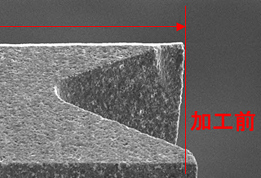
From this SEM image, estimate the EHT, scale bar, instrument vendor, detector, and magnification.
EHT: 10 kV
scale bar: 10000 nm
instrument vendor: JEOL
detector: SEI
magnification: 1.5 K X